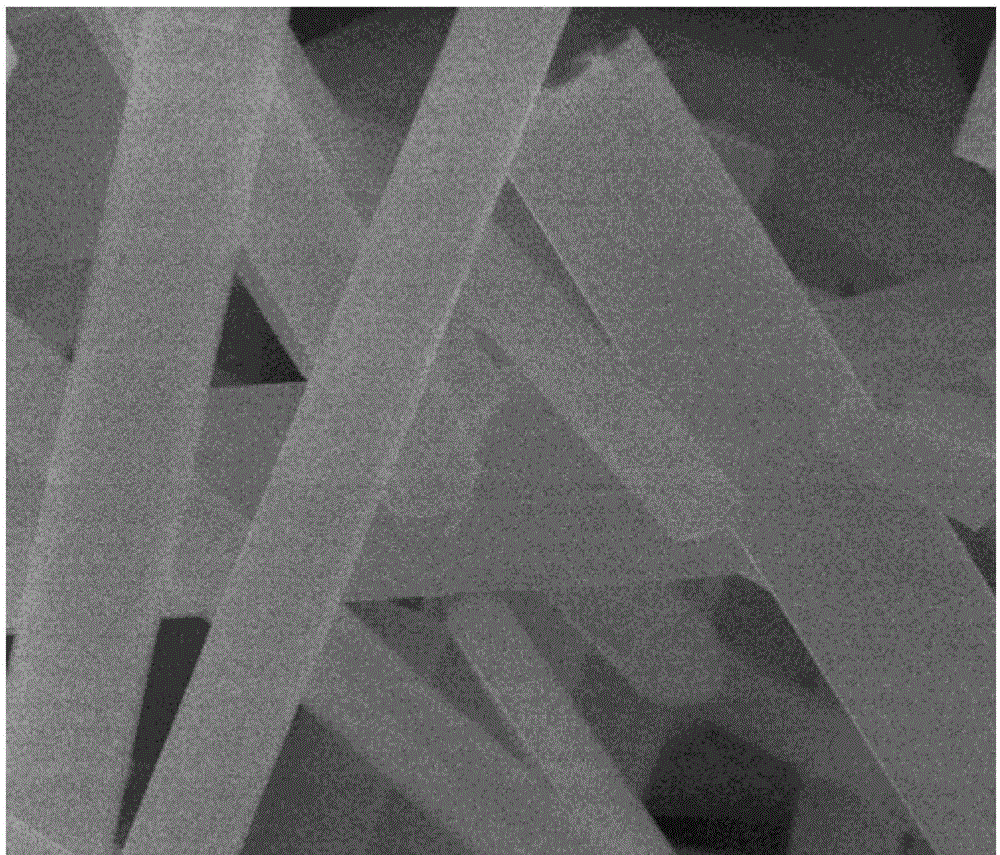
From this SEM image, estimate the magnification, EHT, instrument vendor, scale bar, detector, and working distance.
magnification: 12 K X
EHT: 30 kV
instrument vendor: FEI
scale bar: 5000 nm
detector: ETD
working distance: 9.5 mm